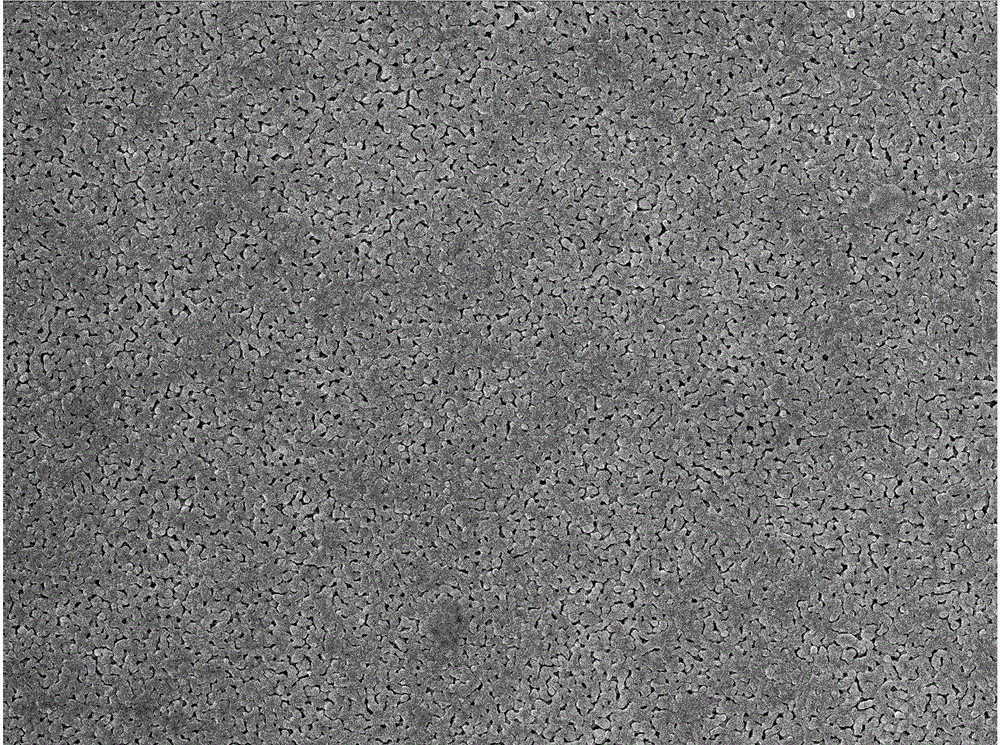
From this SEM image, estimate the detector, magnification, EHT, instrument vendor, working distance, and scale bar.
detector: SEI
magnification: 10 K X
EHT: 10 kV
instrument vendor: JEOL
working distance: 6 mm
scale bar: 1000 nm